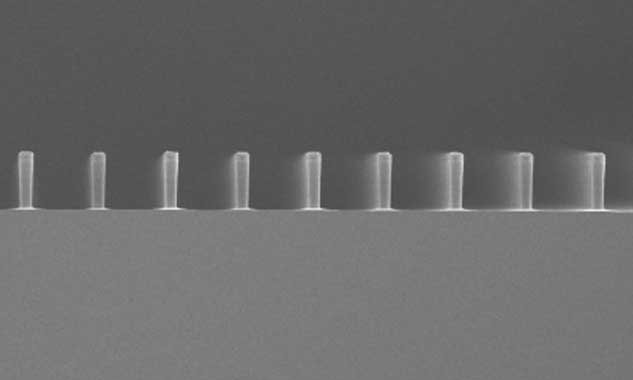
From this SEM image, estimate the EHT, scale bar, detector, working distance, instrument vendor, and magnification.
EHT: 5 kV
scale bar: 100000 nm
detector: SE(UL)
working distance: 8.4 mm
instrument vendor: Hitachi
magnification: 0.4 K X